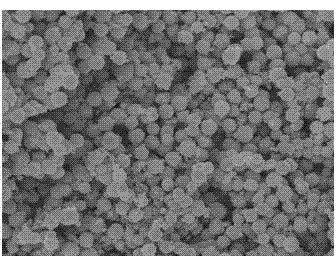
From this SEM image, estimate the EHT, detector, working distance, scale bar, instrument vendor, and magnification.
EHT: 10 kV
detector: SEI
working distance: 8.3 mm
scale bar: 1000 nm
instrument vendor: JEOL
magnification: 10 K X